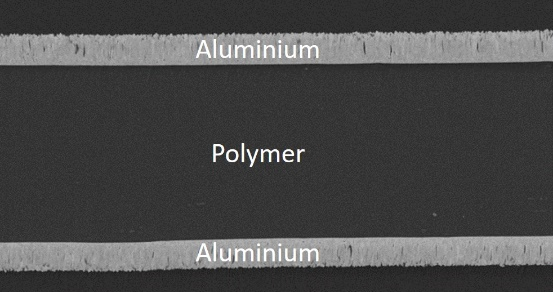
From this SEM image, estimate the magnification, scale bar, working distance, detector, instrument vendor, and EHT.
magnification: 5 K X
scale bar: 10000 nm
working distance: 3.3 mm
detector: LA100(U)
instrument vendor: Hitachi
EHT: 2 kV